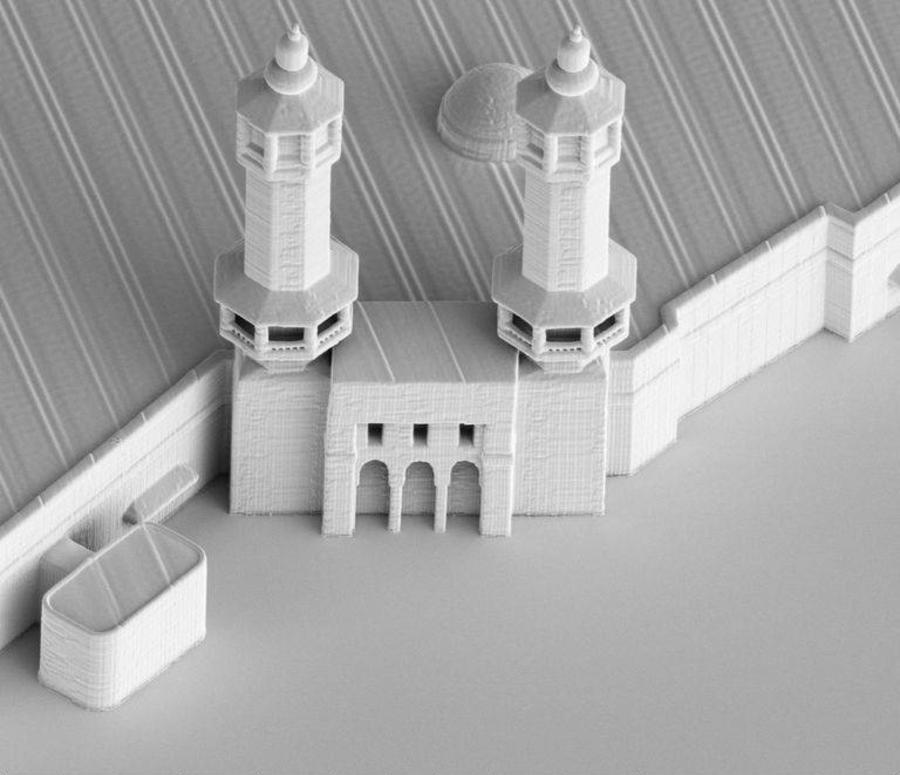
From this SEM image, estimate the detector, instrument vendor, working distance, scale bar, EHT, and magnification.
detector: ETD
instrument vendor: FEI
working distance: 4.3 mm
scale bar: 40000 nm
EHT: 2 kV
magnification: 0.5 K X